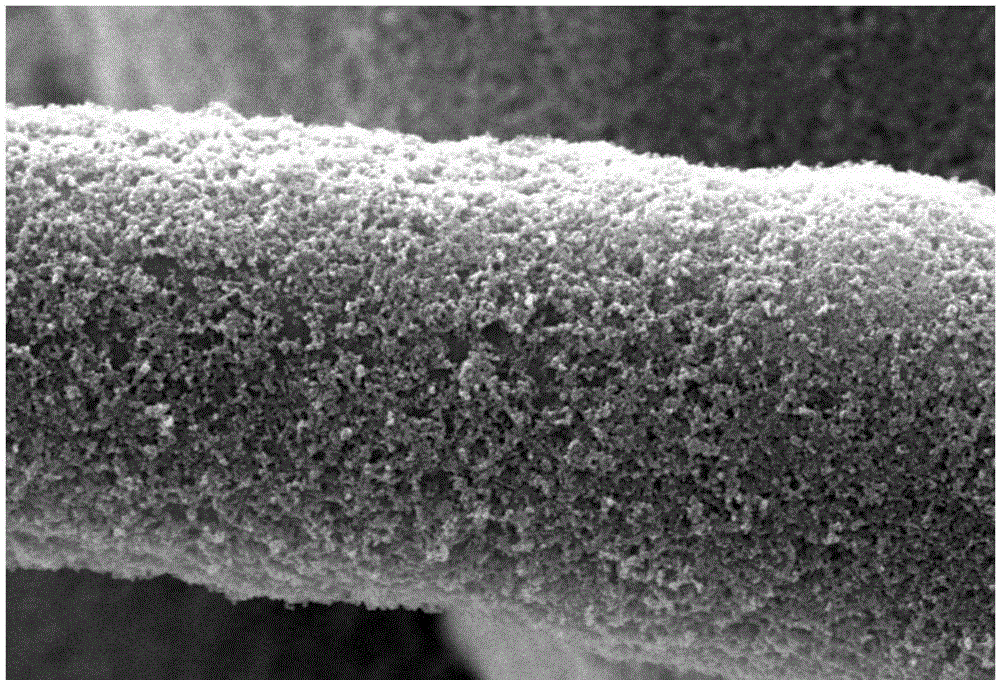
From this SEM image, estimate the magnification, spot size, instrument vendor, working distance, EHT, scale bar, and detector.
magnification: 5 K X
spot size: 40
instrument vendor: JEOL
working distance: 8 mm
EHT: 20 kV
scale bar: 5000 nm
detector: SEI